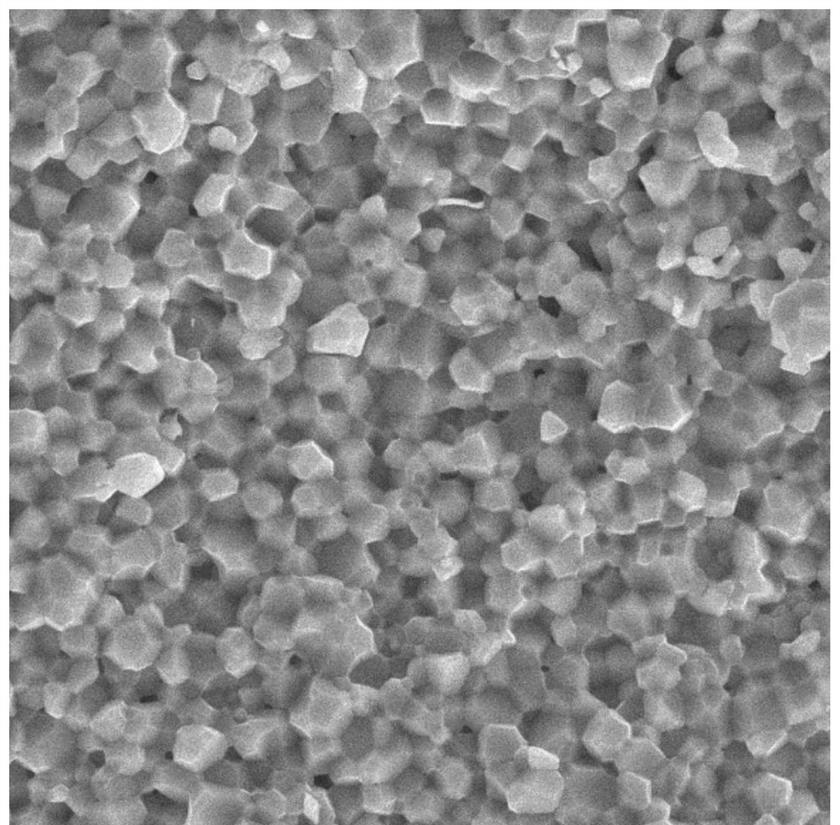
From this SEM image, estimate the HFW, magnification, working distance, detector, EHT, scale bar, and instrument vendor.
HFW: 56.9 µm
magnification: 5 K X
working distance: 10.02 mm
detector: SE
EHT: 30 kV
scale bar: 10000 nm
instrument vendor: Tescan